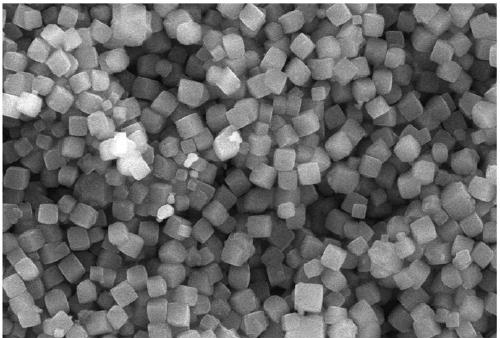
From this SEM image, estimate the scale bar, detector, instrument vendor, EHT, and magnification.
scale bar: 5000 nm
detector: SEI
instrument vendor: JEOL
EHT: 20 kV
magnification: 5 K X